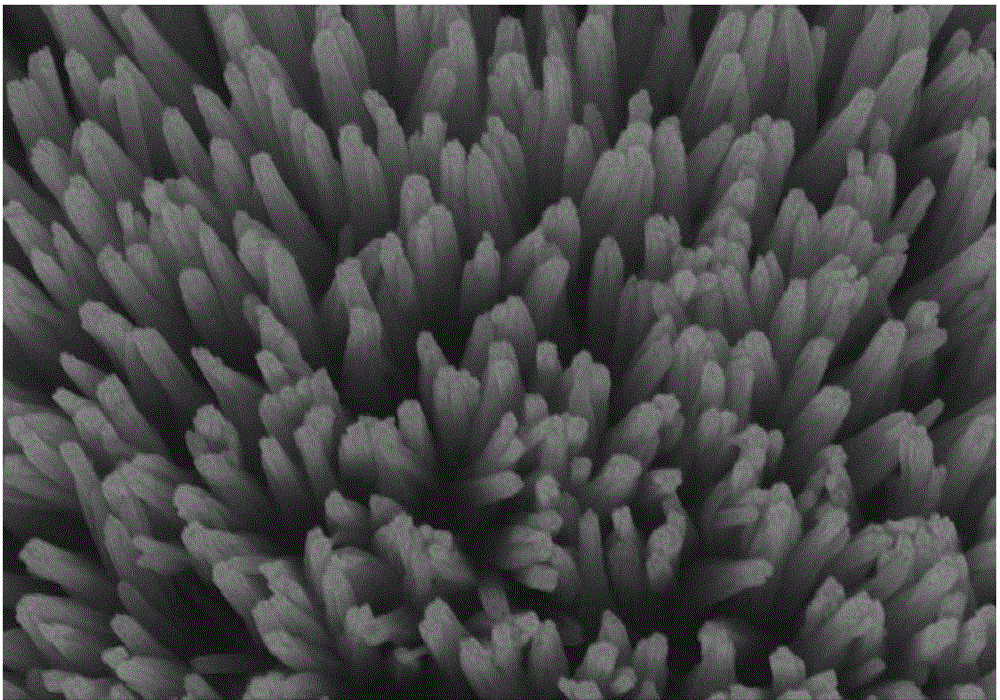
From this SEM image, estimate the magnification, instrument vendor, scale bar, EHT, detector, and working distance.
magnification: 50 K X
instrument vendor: Hitachi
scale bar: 1000 nm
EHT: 10 kV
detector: SE(U,LA0)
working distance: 11.8 mm